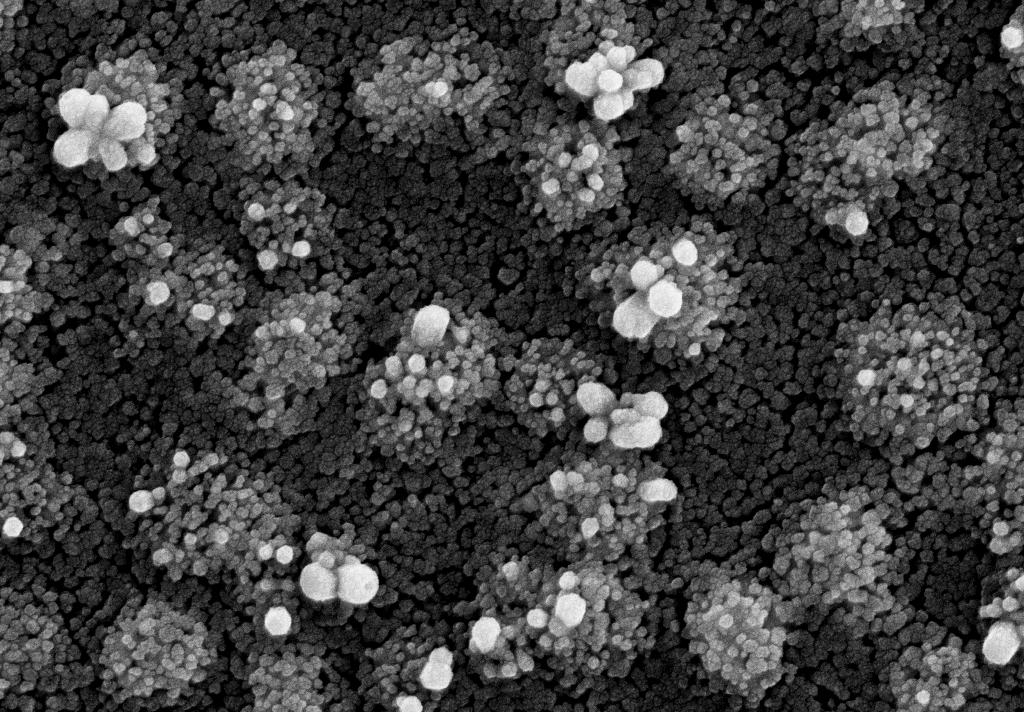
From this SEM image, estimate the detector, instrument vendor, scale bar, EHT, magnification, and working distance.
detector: SE2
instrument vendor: Zeiss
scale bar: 200 nm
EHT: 15 kV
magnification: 50 K X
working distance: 11.4 mm